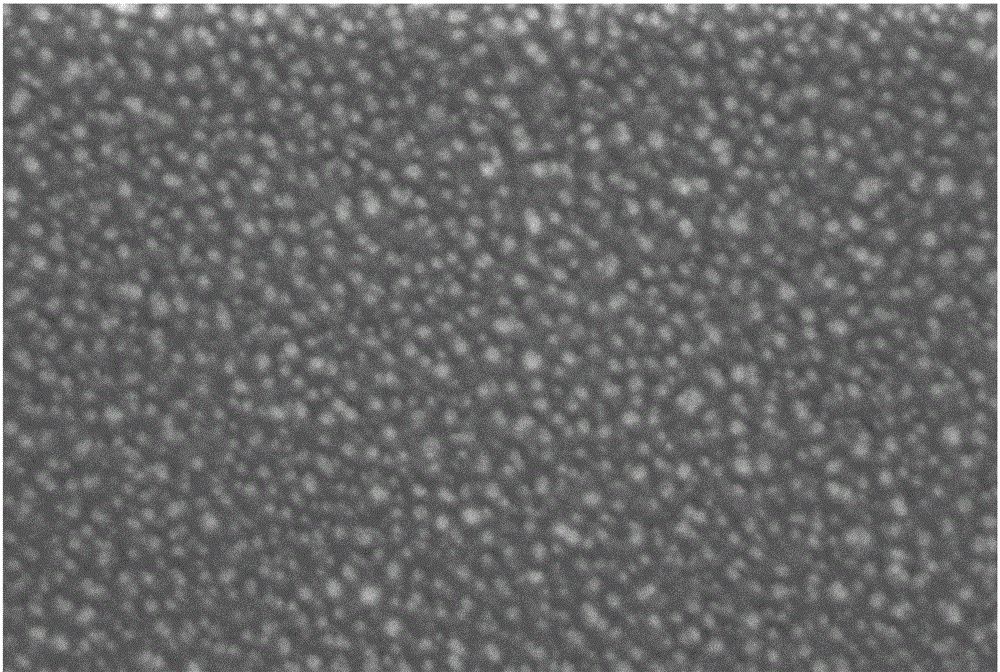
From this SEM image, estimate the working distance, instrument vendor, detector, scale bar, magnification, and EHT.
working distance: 4.8 mm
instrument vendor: Zeiss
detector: InLens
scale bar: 100 nm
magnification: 100 K X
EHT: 5 kV